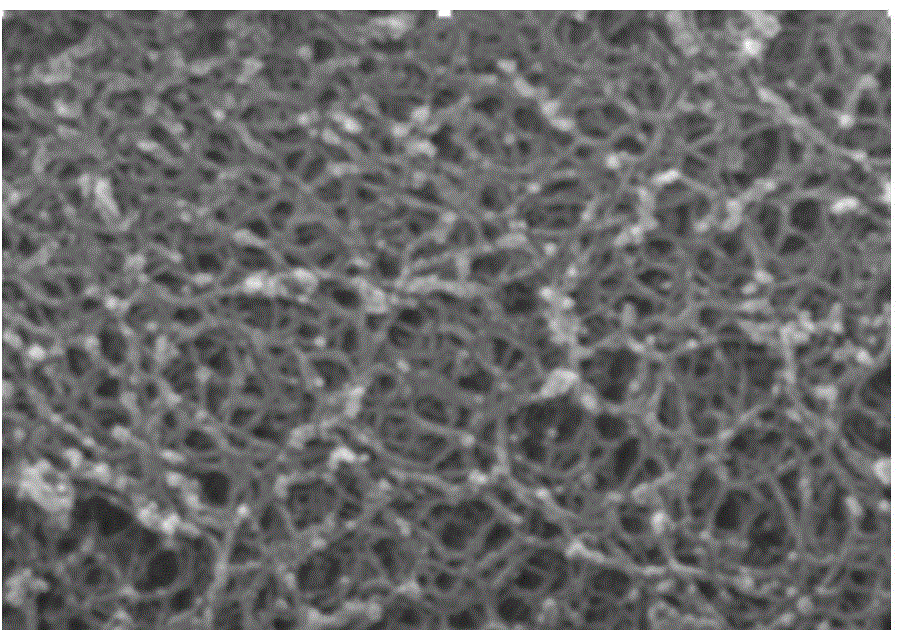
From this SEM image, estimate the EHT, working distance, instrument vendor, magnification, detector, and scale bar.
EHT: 10 kV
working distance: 8.1 mm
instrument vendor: Hitachi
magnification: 100 K X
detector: SE(M)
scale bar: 500 nm